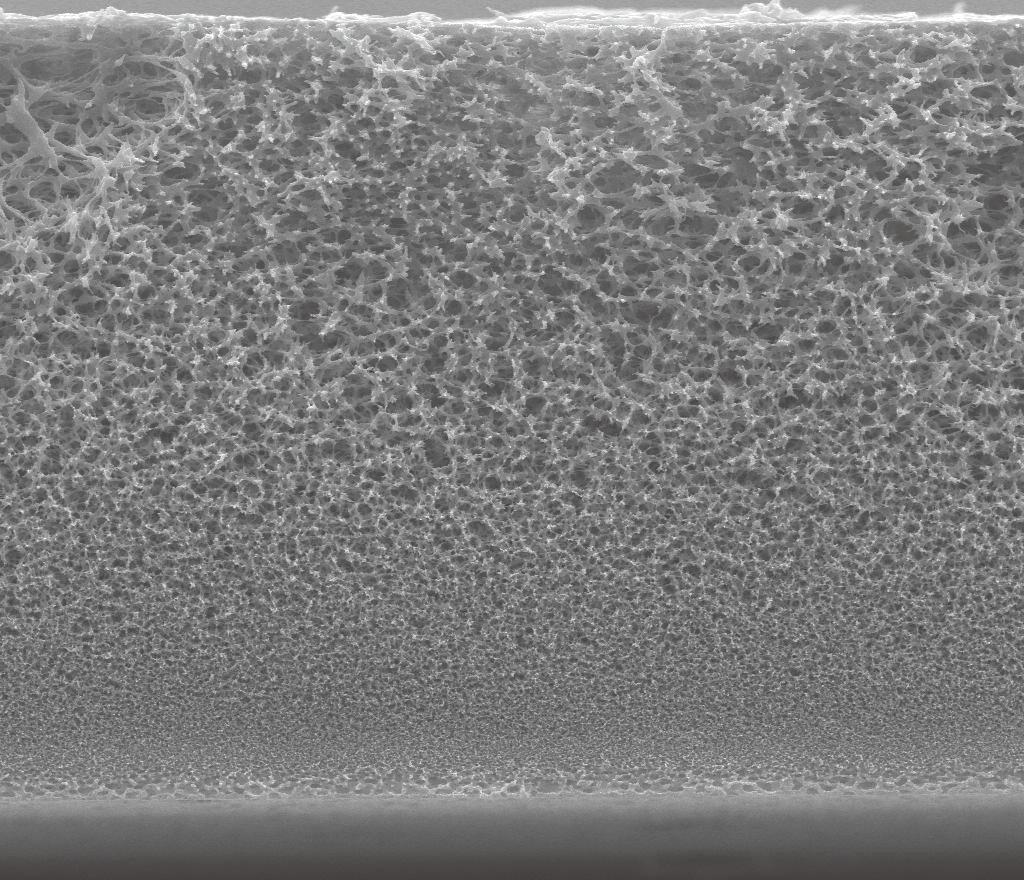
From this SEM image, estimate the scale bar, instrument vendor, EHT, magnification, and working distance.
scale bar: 100000 nm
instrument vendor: FEI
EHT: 15 kV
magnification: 0.8 K X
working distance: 10.7 mm